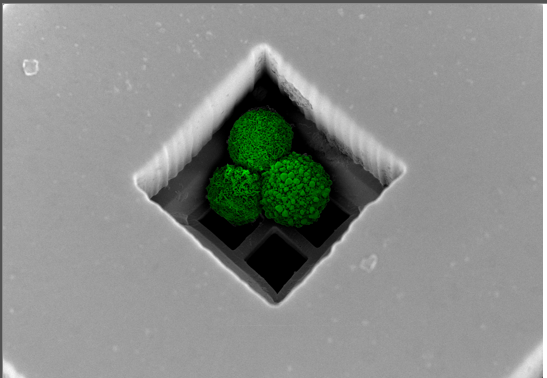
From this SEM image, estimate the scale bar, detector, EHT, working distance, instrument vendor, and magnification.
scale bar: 50000 nm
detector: SE(UL)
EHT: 2 kV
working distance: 5.3 mm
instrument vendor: Hitachi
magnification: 0.9 K X